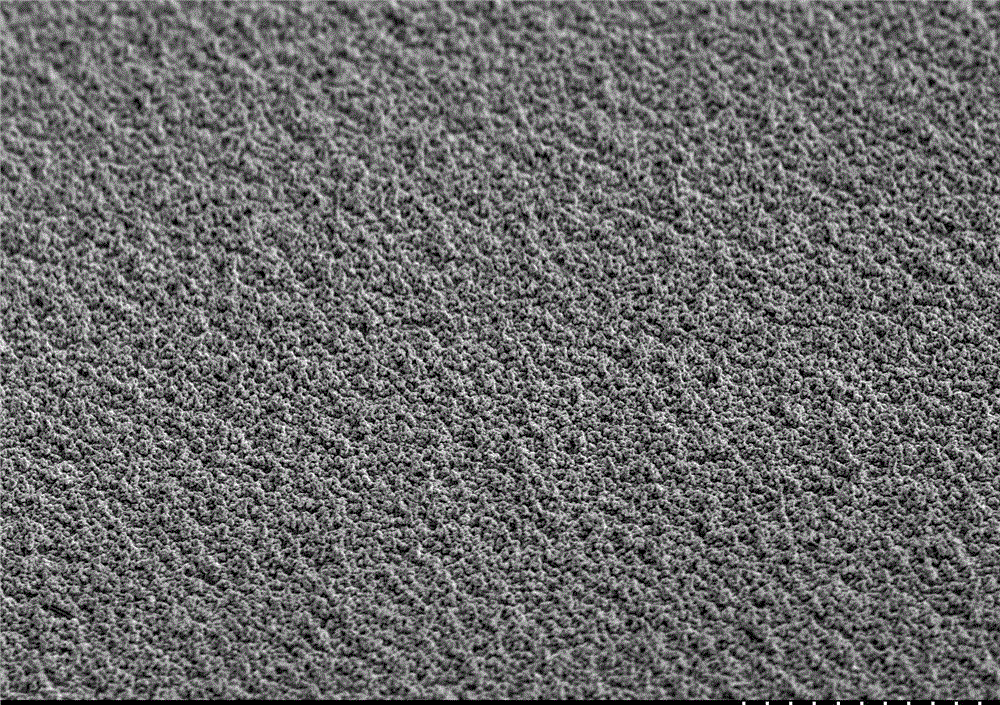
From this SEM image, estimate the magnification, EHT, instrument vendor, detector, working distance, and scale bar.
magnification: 0.3 K X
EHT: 20 kV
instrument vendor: Hitachi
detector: SE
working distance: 19.7 mm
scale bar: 100000 nm